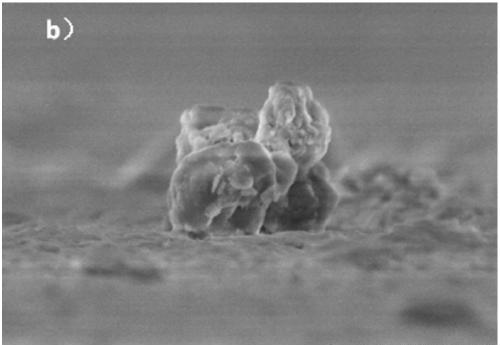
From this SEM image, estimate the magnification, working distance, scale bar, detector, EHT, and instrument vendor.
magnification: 20 K X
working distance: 9.1 mm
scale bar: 2000 nm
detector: SE(UL)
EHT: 3 kV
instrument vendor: Hitachi